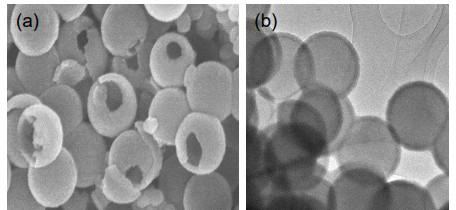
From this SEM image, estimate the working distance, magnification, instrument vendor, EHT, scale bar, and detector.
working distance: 6.5 mm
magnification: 80 K X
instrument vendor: Hitachi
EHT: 15 kV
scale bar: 500 nm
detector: SE(UL)